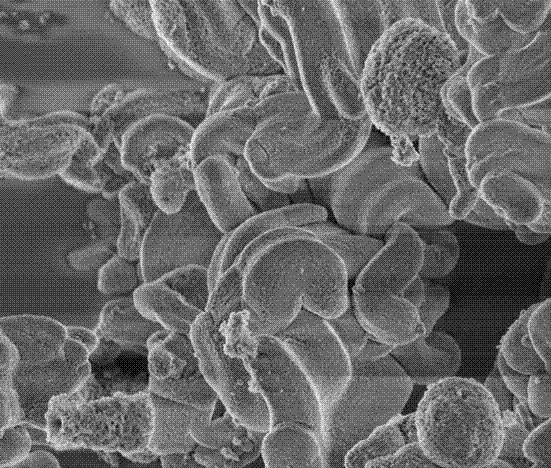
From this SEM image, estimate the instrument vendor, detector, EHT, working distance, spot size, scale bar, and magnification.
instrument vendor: FEI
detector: TLD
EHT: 1 kV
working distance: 2.5 mm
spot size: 3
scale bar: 2000 nm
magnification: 20 K X